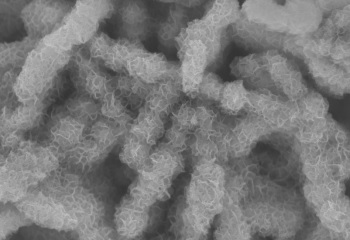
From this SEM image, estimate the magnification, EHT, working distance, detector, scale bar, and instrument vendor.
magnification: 20 K X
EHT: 10 kV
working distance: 7.3 mm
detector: SE(U)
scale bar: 2000 nm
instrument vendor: Hitachi